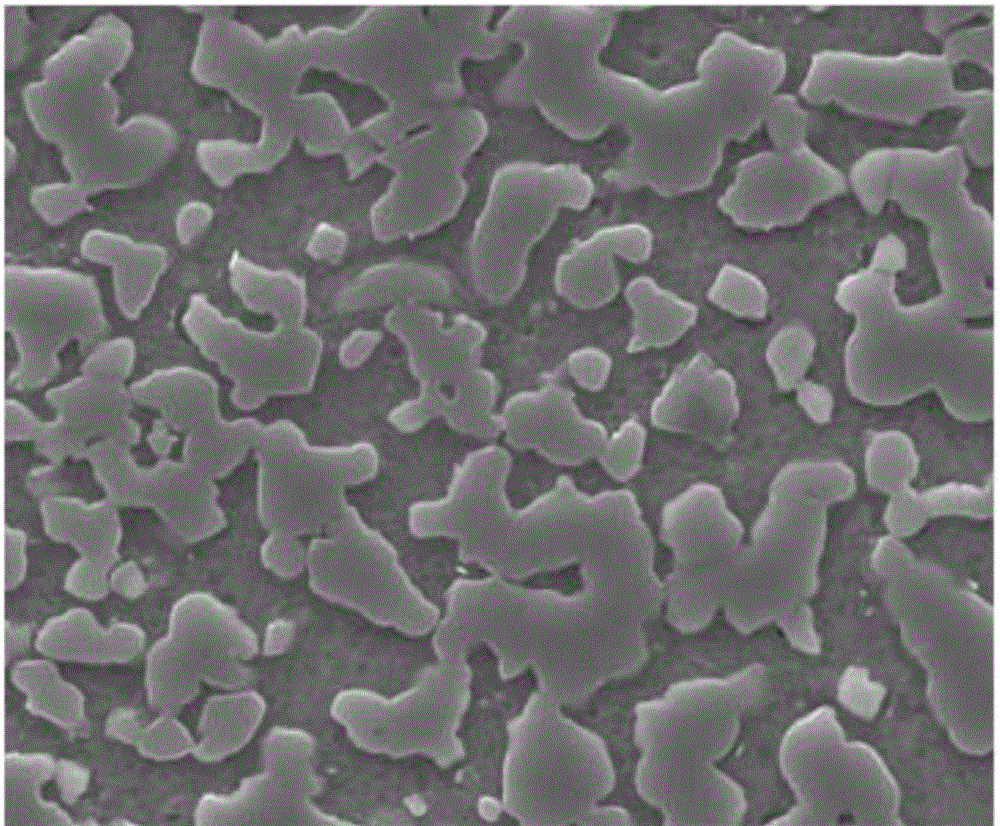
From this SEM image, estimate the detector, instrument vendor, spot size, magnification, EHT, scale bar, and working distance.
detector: ETD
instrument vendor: FEI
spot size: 4.5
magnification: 5 K X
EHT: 20 kV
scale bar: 20000 nm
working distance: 10.5 mm